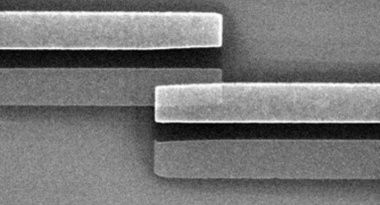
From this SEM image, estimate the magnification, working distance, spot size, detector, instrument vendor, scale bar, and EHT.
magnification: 16.366 K X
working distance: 17 mm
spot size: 3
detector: SE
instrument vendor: FEI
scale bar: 2000 nm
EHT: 10 kV